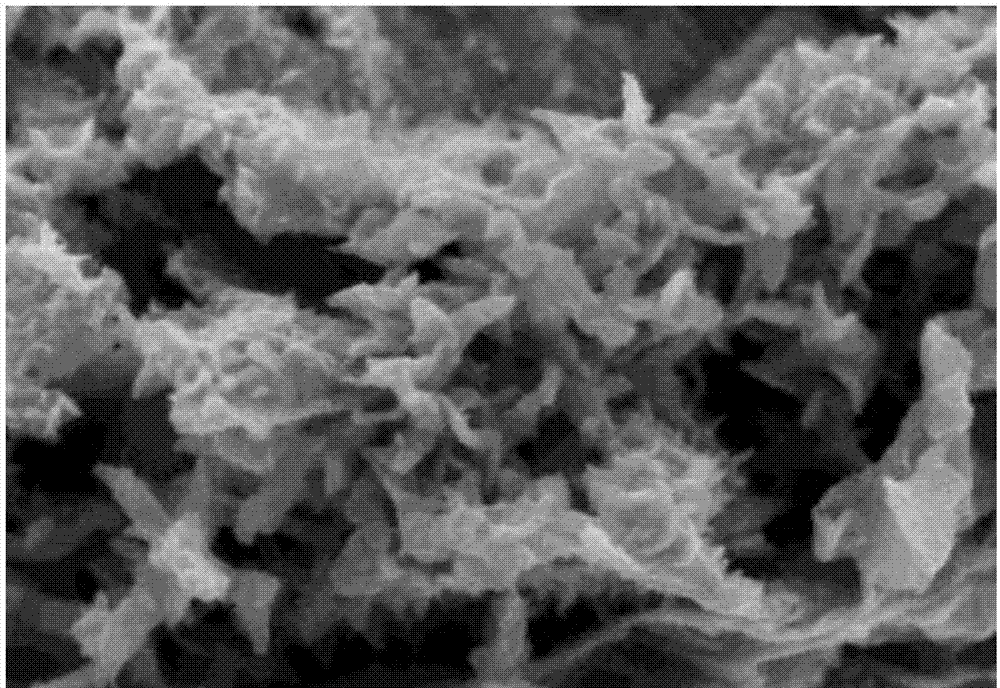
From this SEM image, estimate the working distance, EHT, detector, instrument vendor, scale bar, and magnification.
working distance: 5.8 mm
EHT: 1.5 kV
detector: SE+BSE(U)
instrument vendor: Hitachi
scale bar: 500 nm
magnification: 100 K X